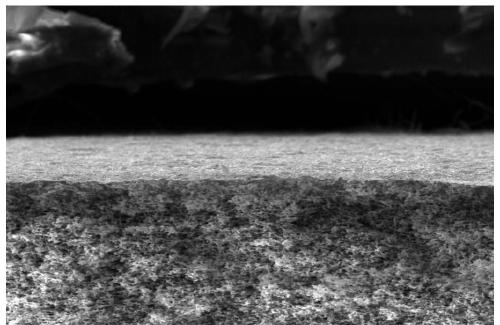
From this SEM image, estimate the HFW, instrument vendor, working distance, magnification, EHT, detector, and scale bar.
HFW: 207 µm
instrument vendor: FEI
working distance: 5.3 mm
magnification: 1 K X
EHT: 18 kV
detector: SE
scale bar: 50000 nm